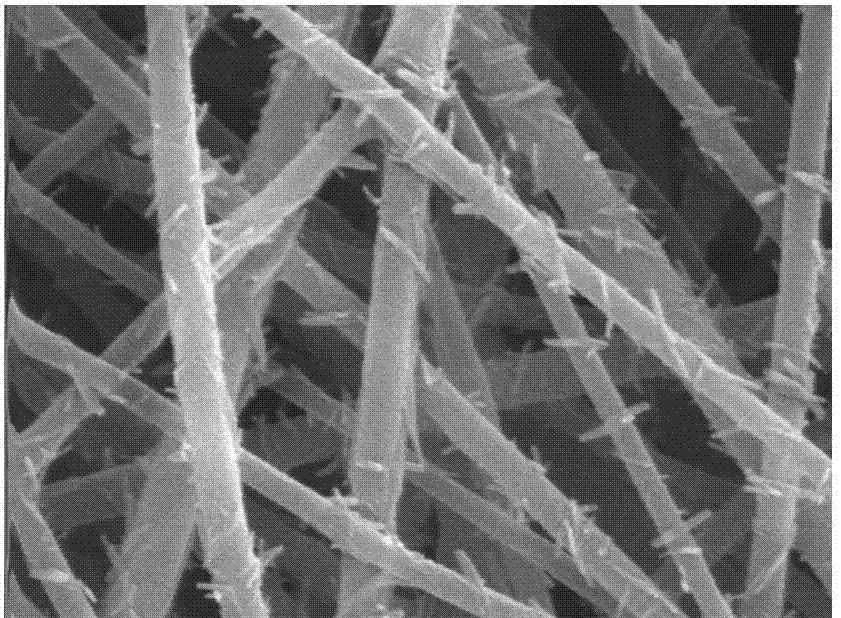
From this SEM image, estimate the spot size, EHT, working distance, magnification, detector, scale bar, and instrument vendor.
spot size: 3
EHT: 15 kV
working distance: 5.9 mm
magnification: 20 K X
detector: SE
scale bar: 1000 nm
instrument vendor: FEI